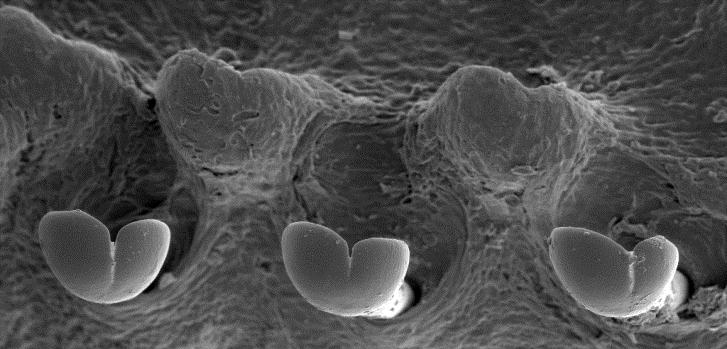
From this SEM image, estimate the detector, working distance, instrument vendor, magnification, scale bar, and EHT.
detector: SE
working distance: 17 mm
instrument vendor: other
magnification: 0.22 K X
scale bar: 50000 nm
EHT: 15 kV